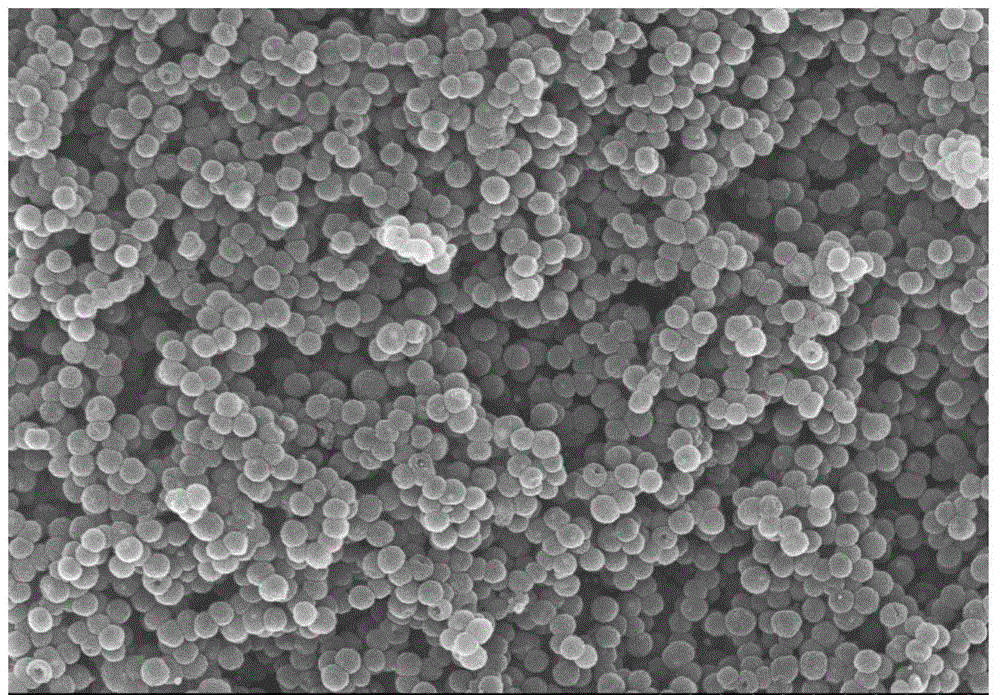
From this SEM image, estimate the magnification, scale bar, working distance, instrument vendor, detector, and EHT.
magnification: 20 K X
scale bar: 2000 nm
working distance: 8.6 mm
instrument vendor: Hitachi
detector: SE(M)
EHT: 10 kV